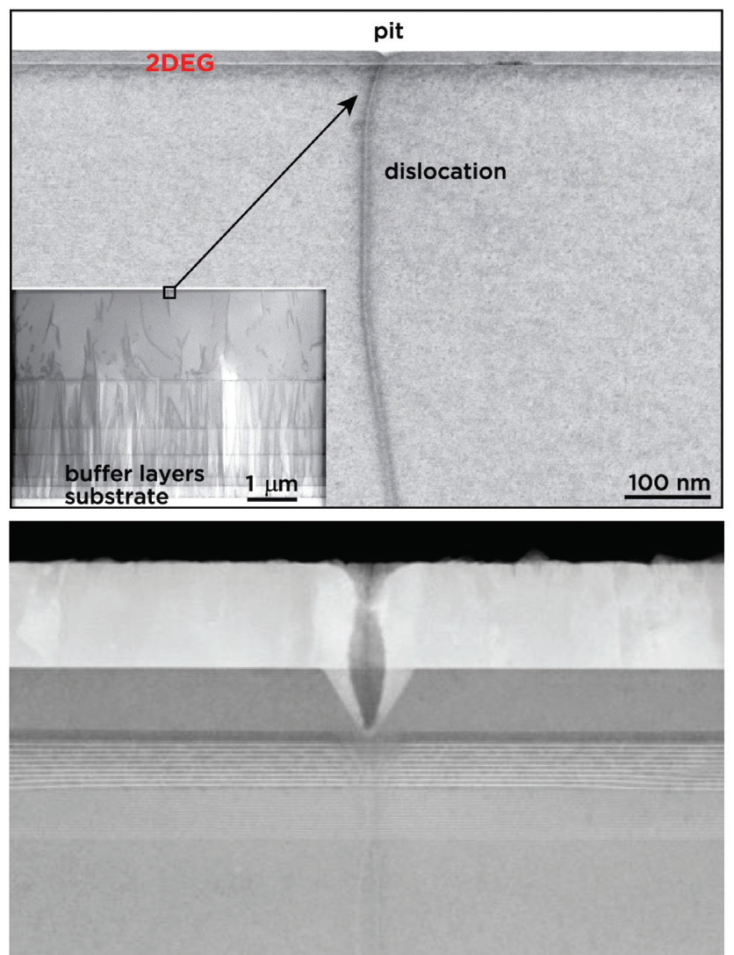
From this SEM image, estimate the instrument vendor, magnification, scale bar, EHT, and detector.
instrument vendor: Hitachi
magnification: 130 K X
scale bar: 200 nm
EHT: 200 kV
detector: ZC